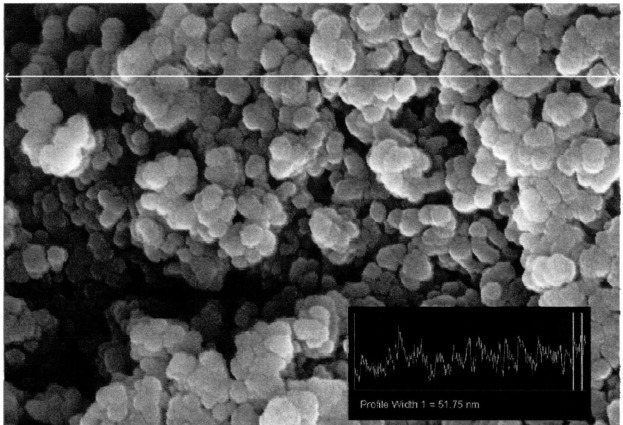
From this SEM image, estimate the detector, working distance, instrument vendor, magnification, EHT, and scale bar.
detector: InLens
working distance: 3.8 mm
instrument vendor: Zeiss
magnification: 199.6 K X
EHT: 5 kV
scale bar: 200 nm